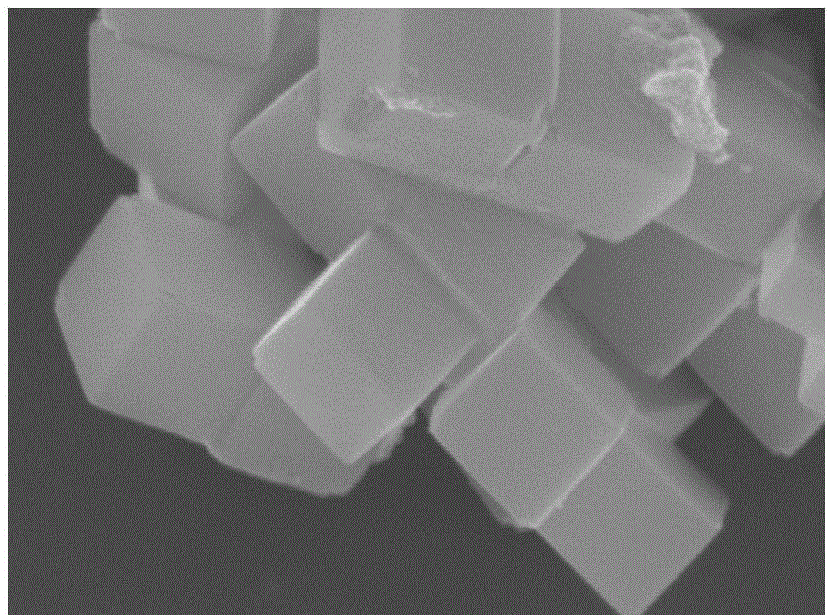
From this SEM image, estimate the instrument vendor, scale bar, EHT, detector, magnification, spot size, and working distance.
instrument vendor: FEI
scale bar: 500 nm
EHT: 10 kV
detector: TLD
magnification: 50 K X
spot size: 3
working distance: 6.6 mm